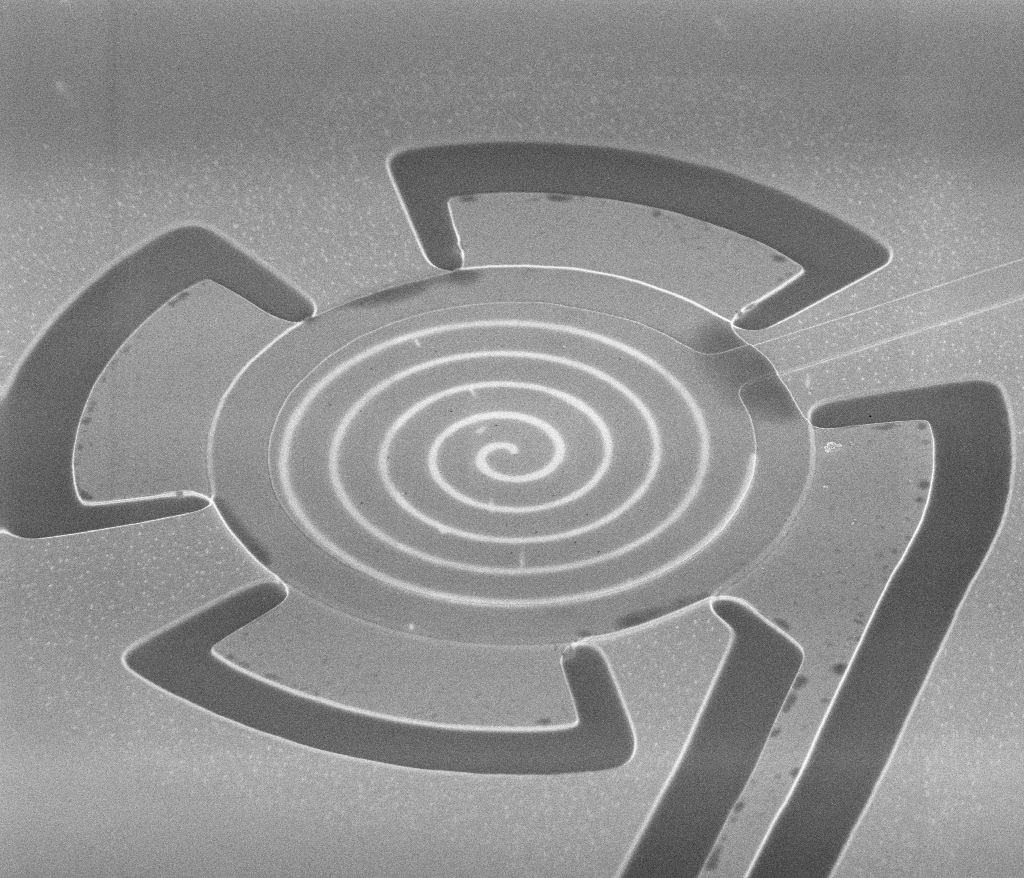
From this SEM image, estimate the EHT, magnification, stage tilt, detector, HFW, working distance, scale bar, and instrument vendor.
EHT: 2 kV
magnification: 5 K X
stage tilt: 52°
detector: TLD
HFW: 51.2 µm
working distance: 4.9 mm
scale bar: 20000 nm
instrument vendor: FEI